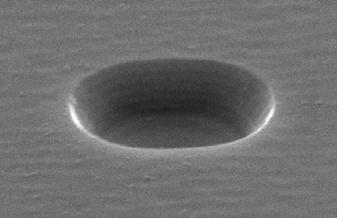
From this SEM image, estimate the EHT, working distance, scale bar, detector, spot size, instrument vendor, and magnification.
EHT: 15 kV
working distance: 18.4 mm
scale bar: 1000 nm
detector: SE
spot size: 2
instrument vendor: FEI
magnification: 17.055 K X